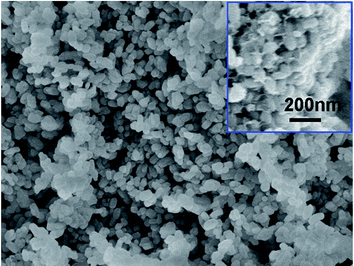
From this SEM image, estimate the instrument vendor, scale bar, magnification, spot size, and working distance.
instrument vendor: FEI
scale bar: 2000 nm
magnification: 80 K X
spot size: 3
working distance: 5.2 mm